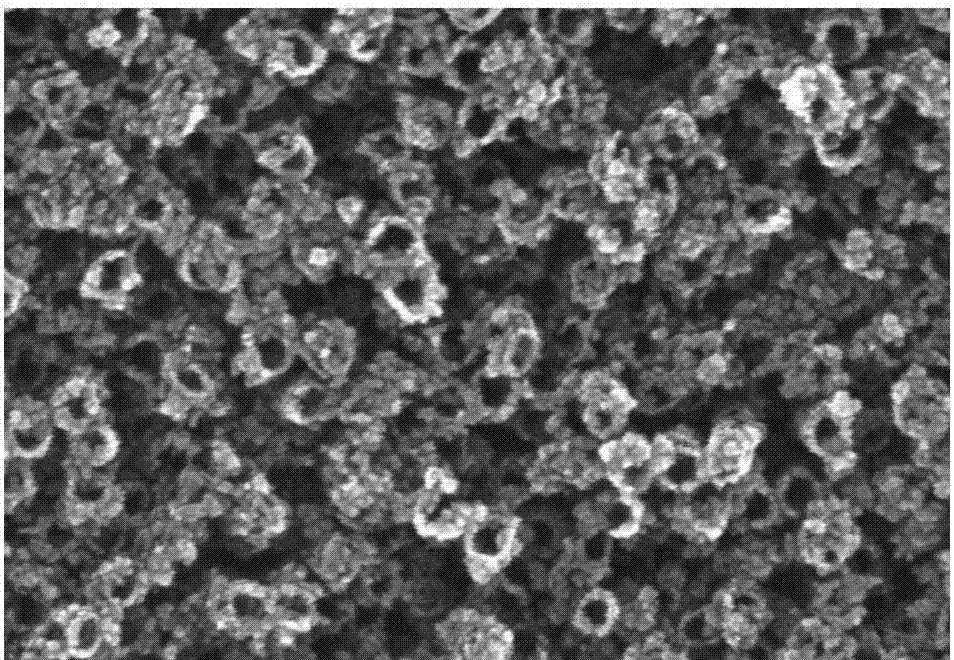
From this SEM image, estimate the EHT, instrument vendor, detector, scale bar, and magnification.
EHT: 20 kV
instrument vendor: Hitachi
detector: SE(U)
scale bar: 500 nm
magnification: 100 K X